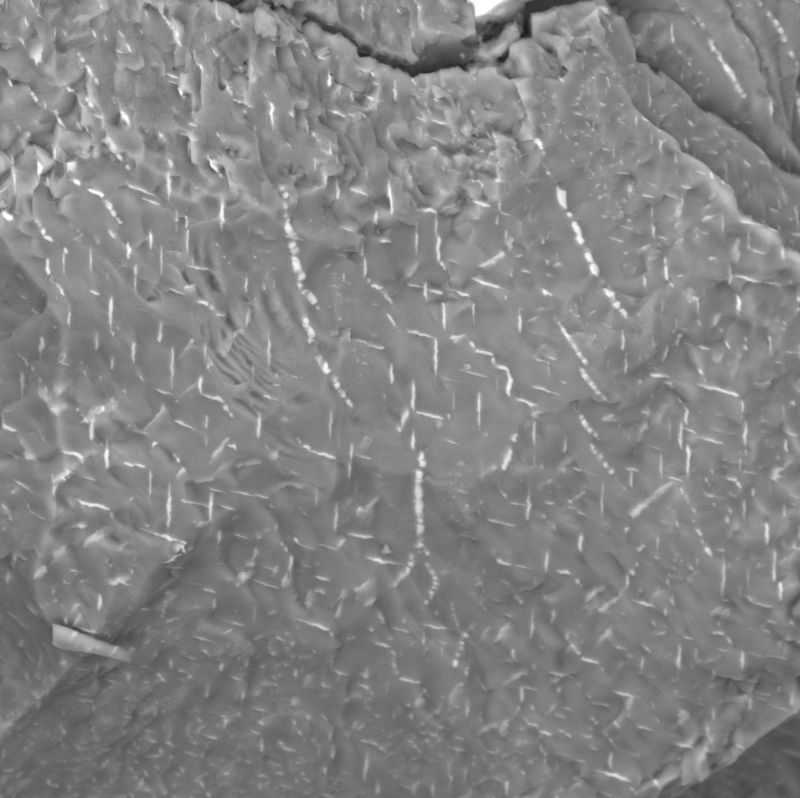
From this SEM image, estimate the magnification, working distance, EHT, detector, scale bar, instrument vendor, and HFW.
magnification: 2.99 K X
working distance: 14.96 mm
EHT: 30 kV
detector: BSE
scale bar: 50000 nm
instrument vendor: Tescan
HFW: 185 µm